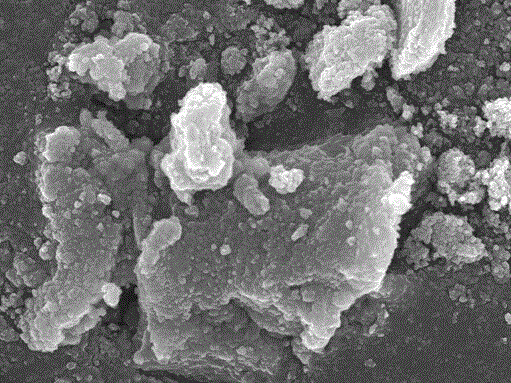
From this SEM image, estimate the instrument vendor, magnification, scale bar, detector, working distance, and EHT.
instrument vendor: JEOL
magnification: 10 K X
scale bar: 1000 nm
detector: SEI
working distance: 2.9 mm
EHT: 10 kV